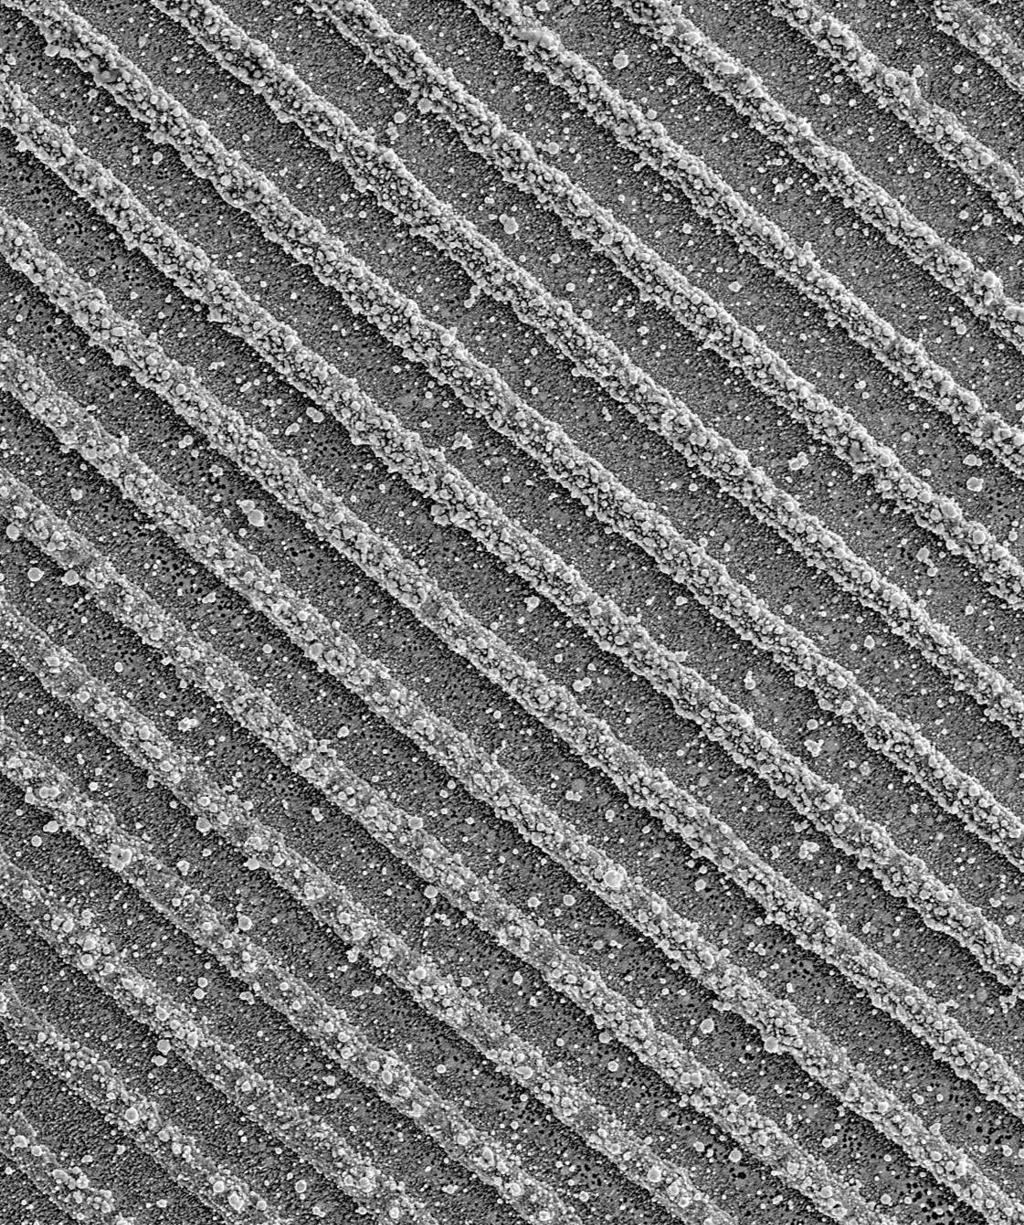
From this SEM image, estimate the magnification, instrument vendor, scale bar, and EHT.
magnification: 2.2 K X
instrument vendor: JEOL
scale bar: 13600 nm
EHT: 5 kV